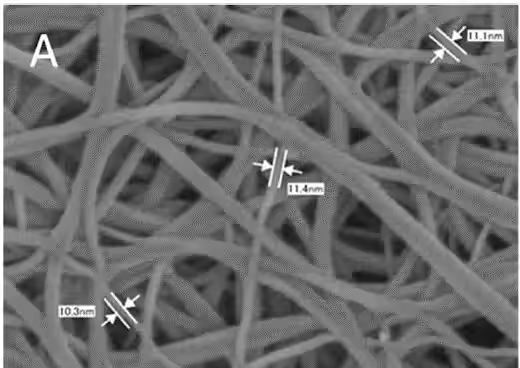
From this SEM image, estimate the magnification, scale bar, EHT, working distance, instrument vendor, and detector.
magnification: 200 K X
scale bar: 100 nm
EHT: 2 kV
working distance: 2 mm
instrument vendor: JEOL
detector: SEI